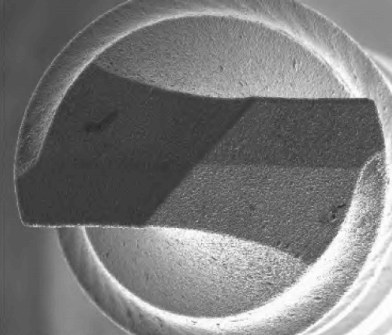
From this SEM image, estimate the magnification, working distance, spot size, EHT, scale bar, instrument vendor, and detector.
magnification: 0.9 K X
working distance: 2.8 mm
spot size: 4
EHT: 10 kV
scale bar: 100000 nm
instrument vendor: FEI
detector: ETD